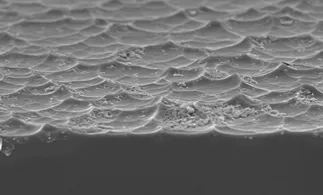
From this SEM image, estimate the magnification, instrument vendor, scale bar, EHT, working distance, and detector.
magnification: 2.5 K X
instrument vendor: Hitachi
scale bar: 20000 nm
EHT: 10 kV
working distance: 8.5 mm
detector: SE(M)